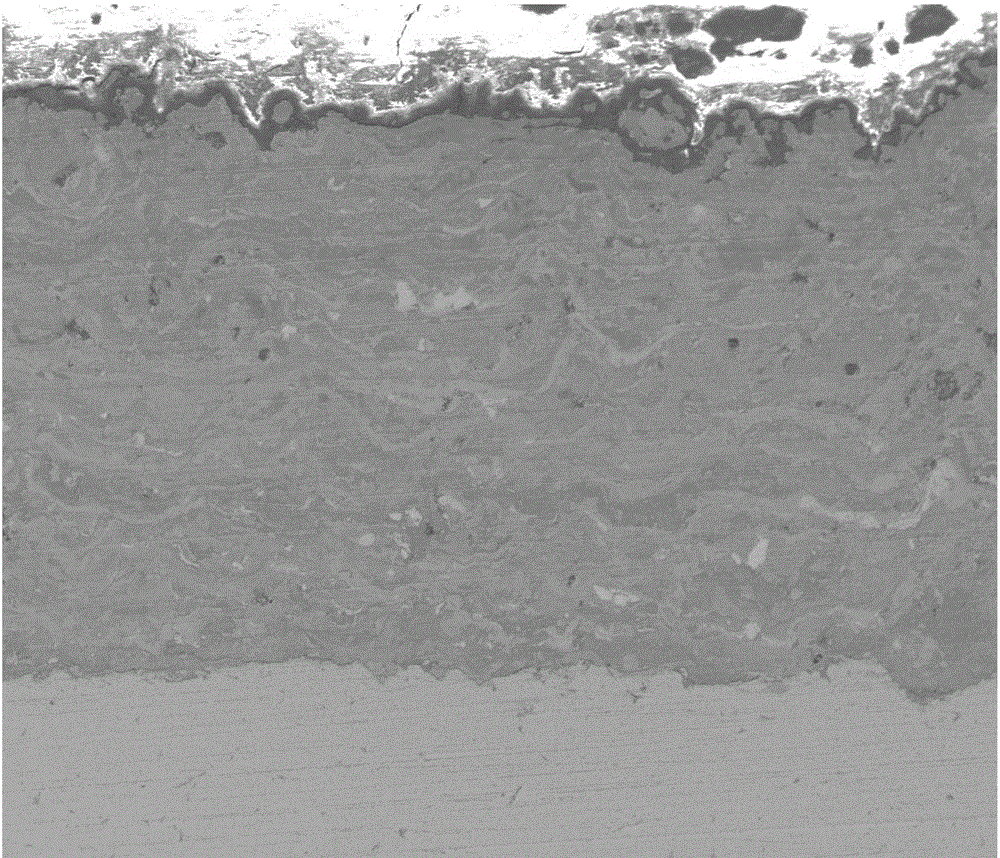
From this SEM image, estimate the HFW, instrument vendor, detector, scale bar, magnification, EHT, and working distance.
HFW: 746 µm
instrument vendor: FEI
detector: ETD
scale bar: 200000 nm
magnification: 0.4 K X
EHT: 5 kV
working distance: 9.1 mm